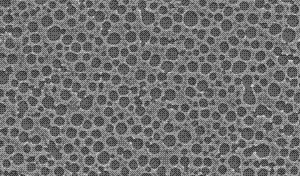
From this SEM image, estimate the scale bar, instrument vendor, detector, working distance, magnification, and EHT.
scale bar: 1000 nm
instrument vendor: Zeiss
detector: SE2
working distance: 7 mm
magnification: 5.06 K X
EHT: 10 kV